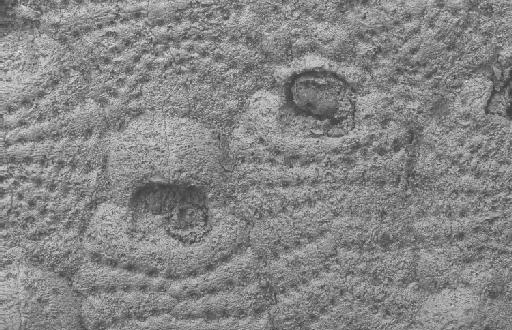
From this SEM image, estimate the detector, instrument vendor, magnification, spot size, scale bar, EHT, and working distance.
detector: QBSD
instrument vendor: Zeiss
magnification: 0.35 K X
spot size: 500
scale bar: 100000 nm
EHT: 15 kV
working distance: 12 mm